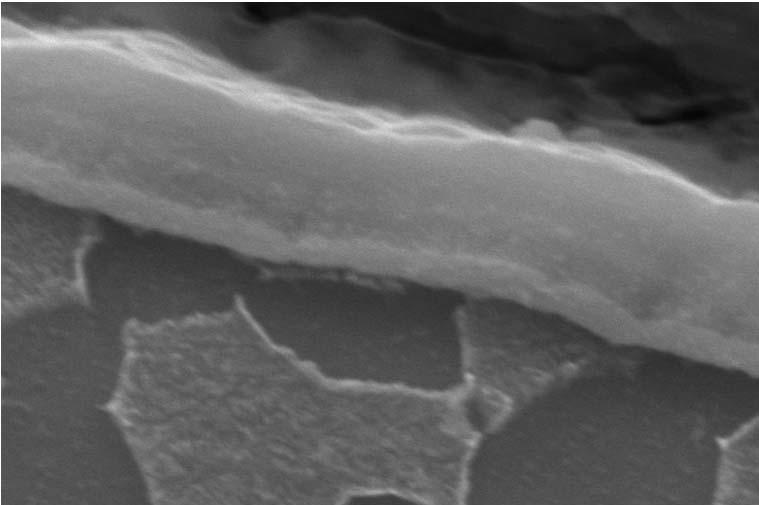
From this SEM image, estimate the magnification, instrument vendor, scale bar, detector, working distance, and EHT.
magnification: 30 K X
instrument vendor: Zeiss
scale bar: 1000 nm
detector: SE1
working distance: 8.5 mm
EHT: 20 kV